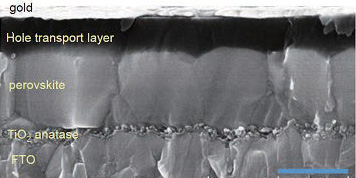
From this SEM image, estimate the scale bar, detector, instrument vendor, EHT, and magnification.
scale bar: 1000 nm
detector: SE(U)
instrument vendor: Hitachi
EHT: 10 kV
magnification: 50 K X